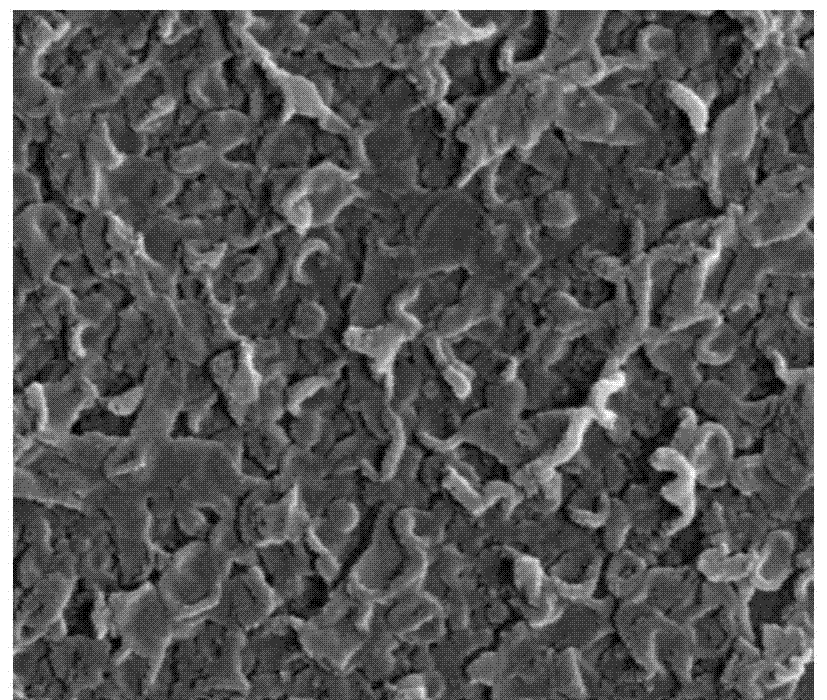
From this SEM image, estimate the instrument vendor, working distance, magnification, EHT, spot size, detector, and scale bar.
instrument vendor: FEI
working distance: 5 mm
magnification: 80 K X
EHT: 10 kV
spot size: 3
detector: TLD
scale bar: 500 nm